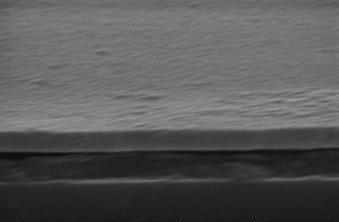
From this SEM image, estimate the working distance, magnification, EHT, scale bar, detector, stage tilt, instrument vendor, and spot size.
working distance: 15.7 mm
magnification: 53.482 K X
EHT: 20 kV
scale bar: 500 nm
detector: SE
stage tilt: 0°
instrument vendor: FEI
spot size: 3